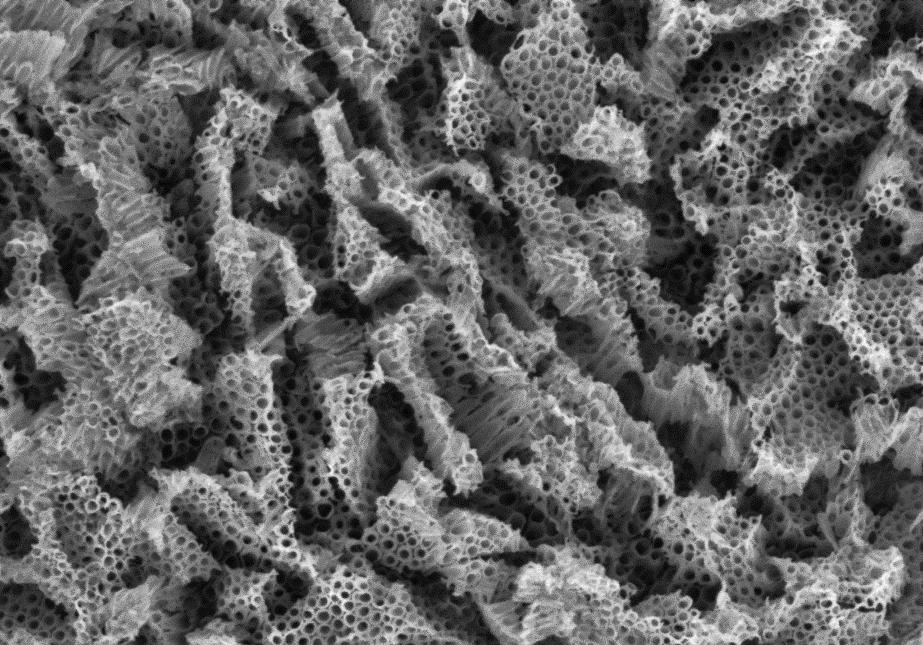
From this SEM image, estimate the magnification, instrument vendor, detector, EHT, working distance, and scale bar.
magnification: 50 K X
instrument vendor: Zeiss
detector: InLens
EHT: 3 kV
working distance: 5.7 mm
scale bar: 200 nm